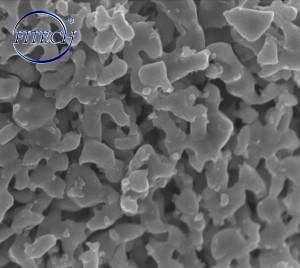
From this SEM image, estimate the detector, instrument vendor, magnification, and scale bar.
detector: SE(U)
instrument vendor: Hitachi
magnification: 50 K X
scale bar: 1000 nm